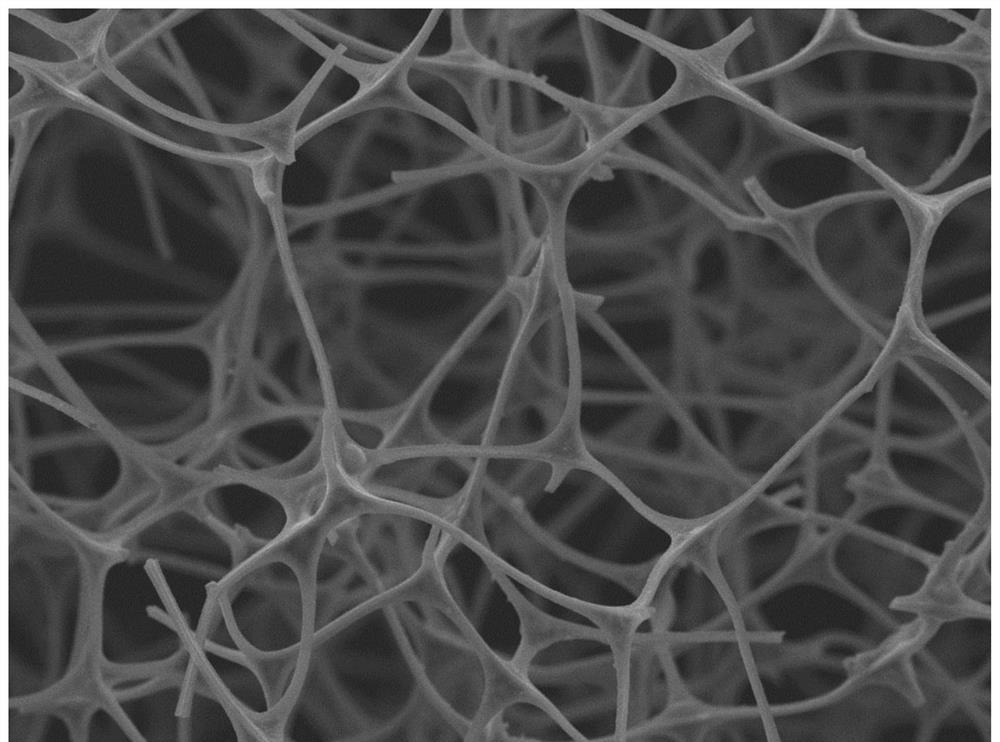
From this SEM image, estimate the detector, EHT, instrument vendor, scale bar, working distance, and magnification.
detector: SEI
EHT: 15 kV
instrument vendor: JEOL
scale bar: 10000 nm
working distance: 6 mm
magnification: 0.5 K X